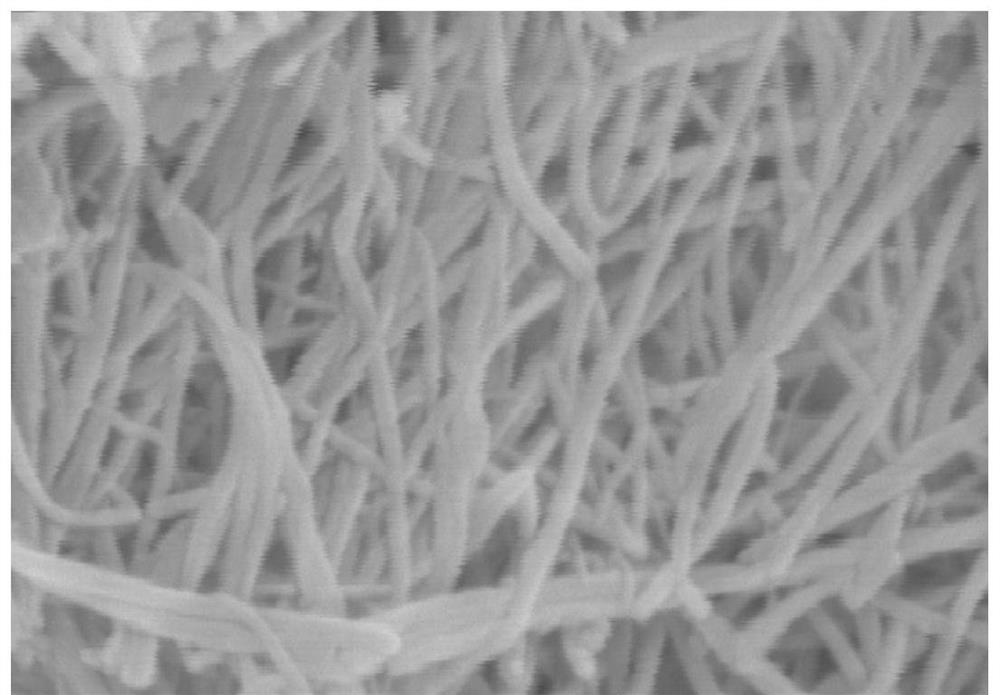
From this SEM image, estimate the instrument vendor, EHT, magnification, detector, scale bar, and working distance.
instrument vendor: Hitachi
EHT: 15 kV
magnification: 100 K X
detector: SE(M)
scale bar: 500 nm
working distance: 11.8 mm